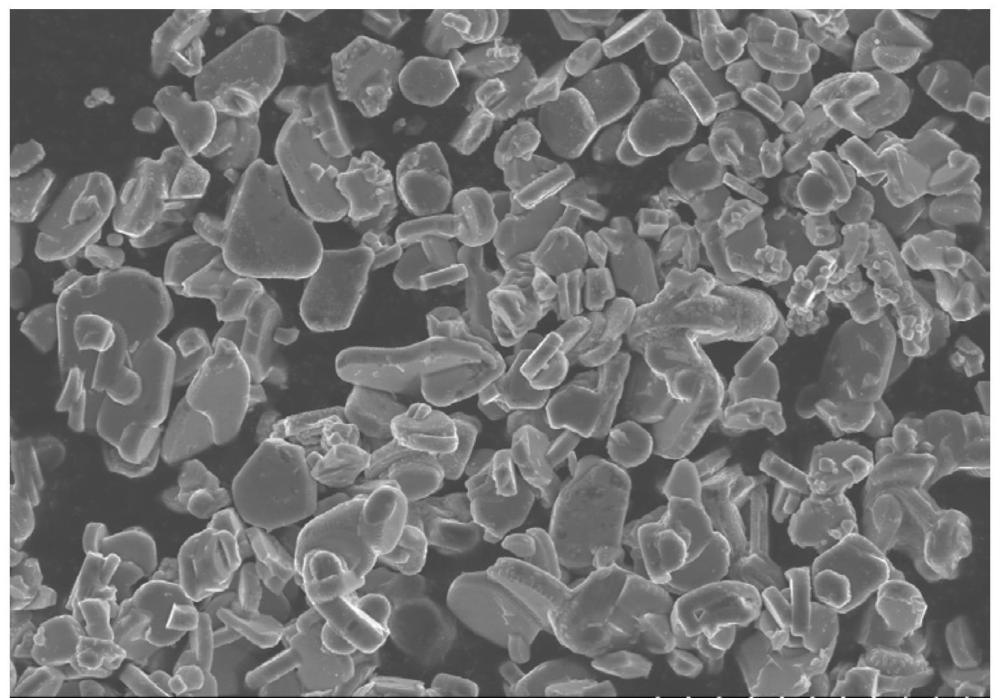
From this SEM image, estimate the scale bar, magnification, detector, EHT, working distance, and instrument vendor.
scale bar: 20000 nm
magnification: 2 K X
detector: SE(UL)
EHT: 10 kV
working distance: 8.2 mm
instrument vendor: Hitachi